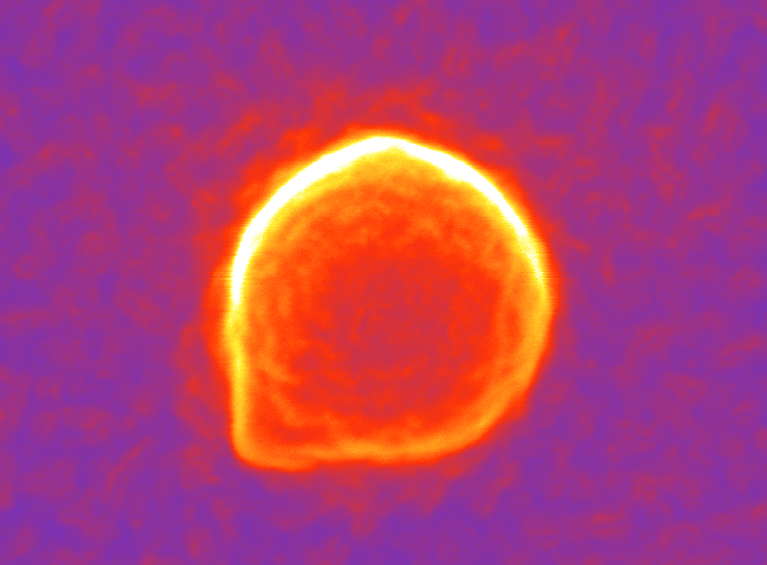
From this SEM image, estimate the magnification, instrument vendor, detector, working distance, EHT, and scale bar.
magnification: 150 K X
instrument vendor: JEOL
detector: SED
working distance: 8.5 mm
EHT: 10 kV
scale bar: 100 nm